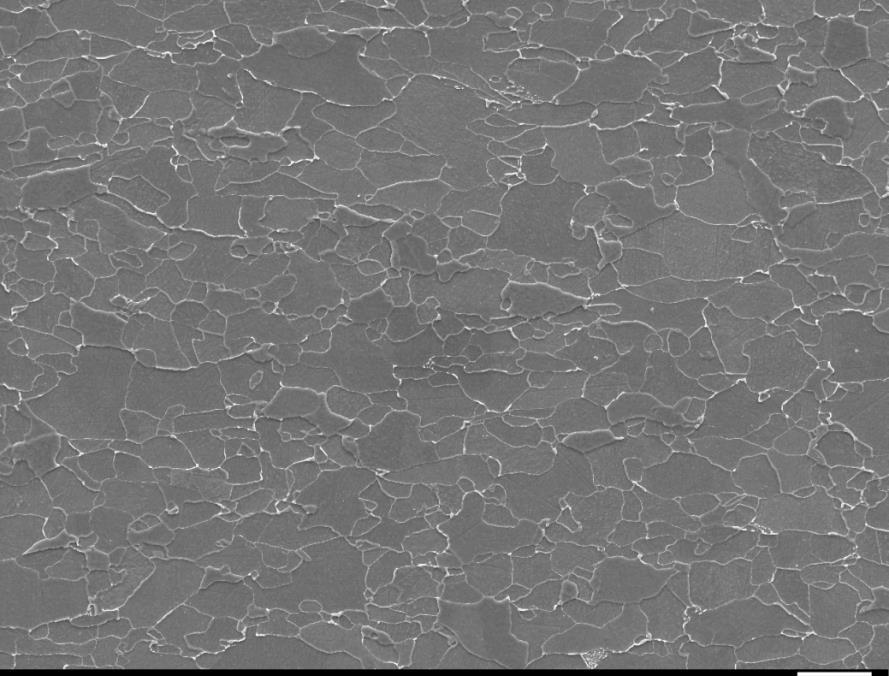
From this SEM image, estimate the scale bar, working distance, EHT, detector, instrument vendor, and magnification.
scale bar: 10000 nm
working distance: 9.8 mm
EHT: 15 kV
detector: SEI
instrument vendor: JEOL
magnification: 1 K X